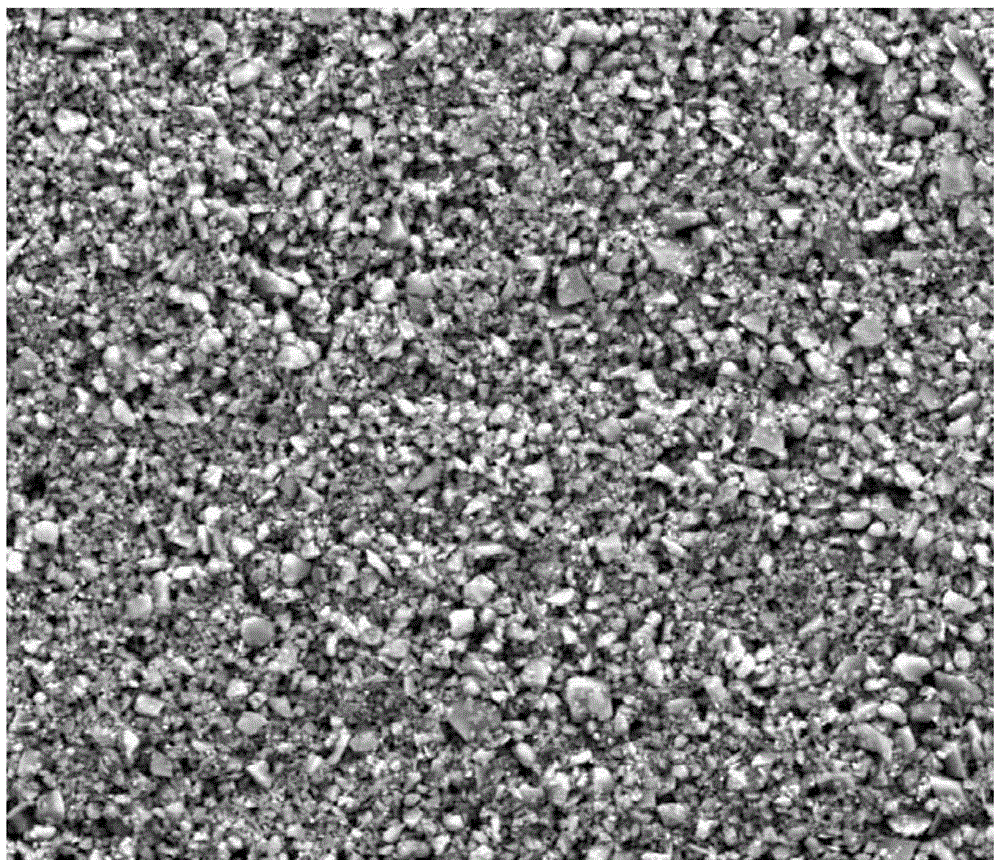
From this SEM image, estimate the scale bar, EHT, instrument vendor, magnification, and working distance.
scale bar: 50000 nm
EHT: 20 kV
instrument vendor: FEI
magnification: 2 K X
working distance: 17.1 mm